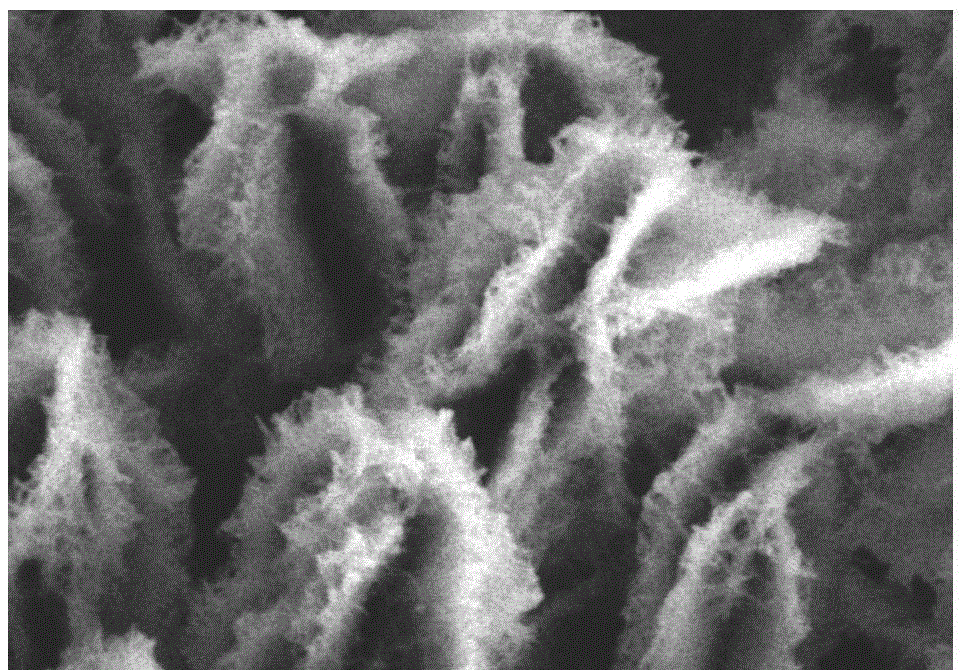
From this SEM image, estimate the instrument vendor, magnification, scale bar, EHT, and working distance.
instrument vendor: Hitachi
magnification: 40 K X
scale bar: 1000 nm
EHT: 3 kV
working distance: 13.4 mm